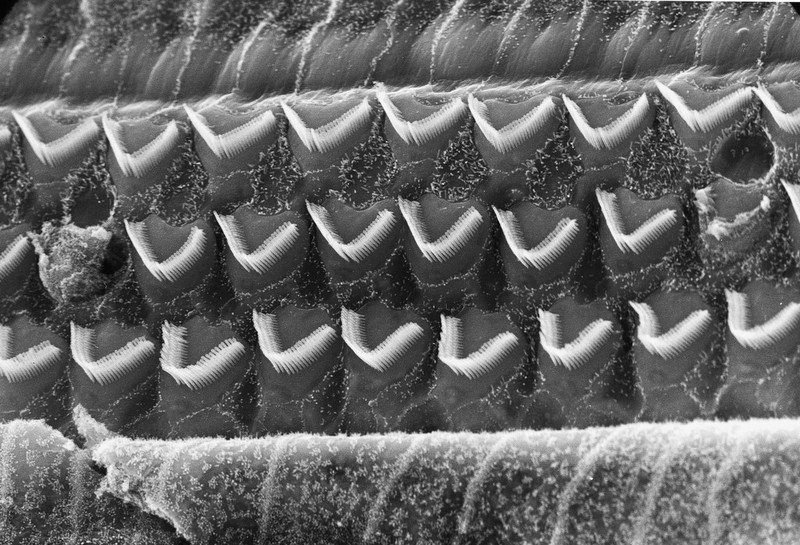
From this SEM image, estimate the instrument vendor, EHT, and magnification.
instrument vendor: JEOL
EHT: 25 kV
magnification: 2 K X